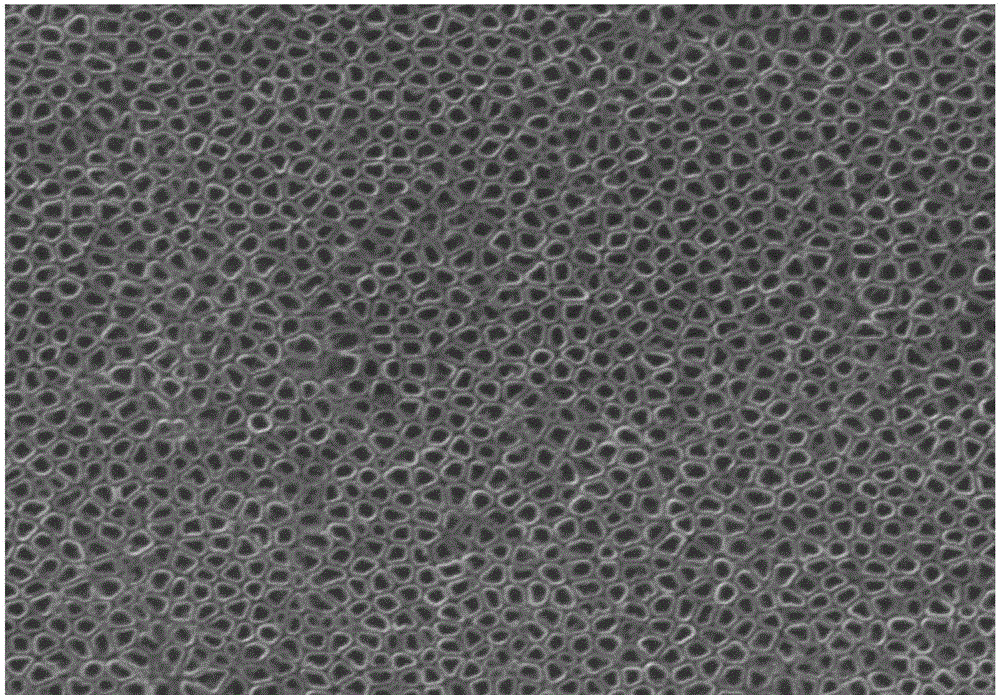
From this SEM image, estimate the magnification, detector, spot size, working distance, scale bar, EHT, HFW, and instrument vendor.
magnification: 60 K X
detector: ETD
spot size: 2.5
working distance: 10.2 mm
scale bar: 1000 nm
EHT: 30 kV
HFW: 4.97 µm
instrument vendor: FEI